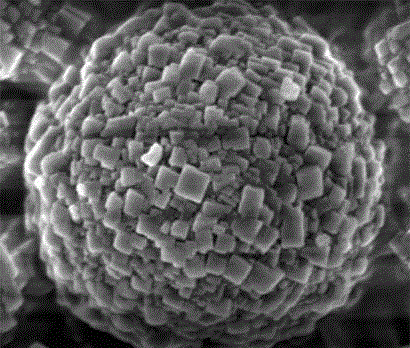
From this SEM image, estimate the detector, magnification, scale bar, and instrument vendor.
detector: TLD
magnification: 80 K X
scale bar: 500 nm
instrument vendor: FEI